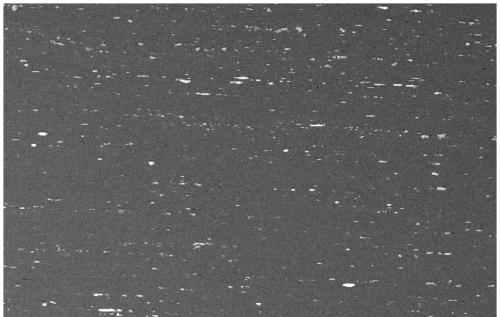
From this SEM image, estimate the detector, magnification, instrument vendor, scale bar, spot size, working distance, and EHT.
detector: BSE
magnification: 0.5 K X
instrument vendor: FEI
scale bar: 100000 nm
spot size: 4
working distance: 7 mm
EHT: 20 kV